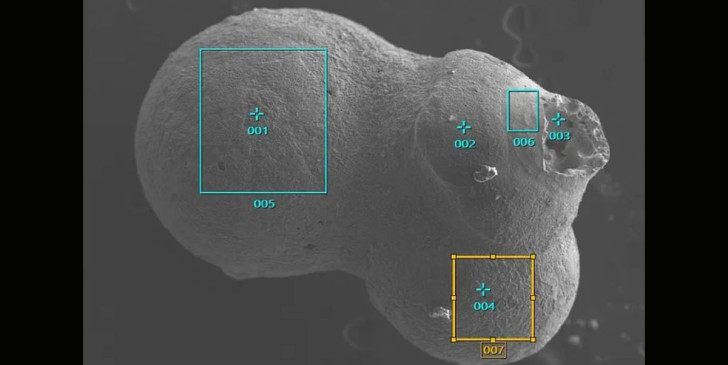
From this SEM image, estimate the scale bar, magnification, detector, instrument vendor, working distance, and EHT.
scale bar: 100000 nm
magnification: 0.07 K X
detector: SEI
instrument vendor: JEOL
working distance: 11.1 mm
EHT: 15 kV